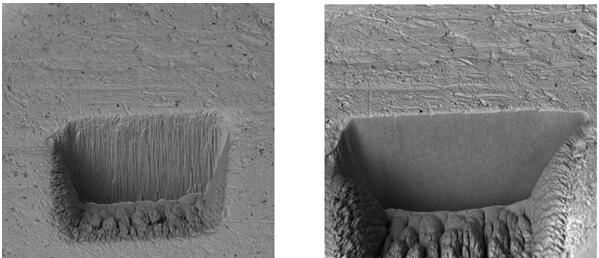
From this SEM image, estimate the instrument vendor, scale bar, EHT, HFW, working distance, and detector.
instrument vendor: Tescan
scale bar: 50000 nm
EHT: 10 kV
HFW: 190 µm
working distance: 9.08 mm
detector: BSE